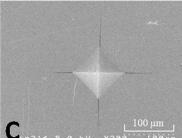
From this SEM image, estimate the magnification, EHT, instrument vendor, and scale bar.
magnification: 0.3 K X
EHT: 5 kV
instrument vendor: JEOL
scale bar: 100000 nm